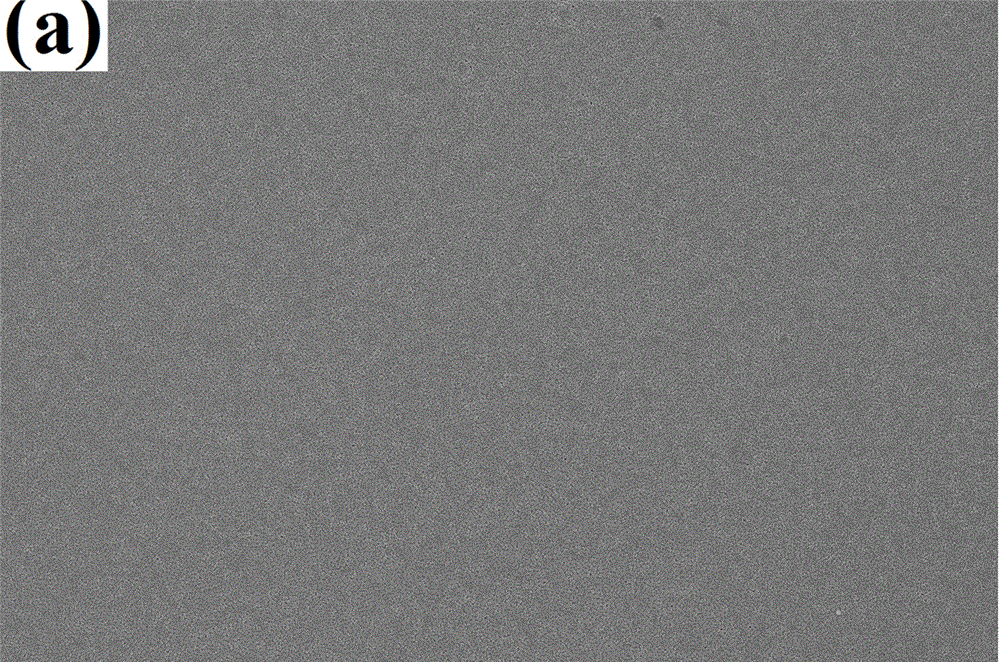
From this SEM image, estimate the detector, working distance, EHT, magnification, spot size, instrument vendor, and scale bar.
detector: TLD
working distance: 4.8 mm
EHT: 5 kV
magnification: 10 K X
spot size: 3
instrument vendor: FEI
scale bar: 10000 nm